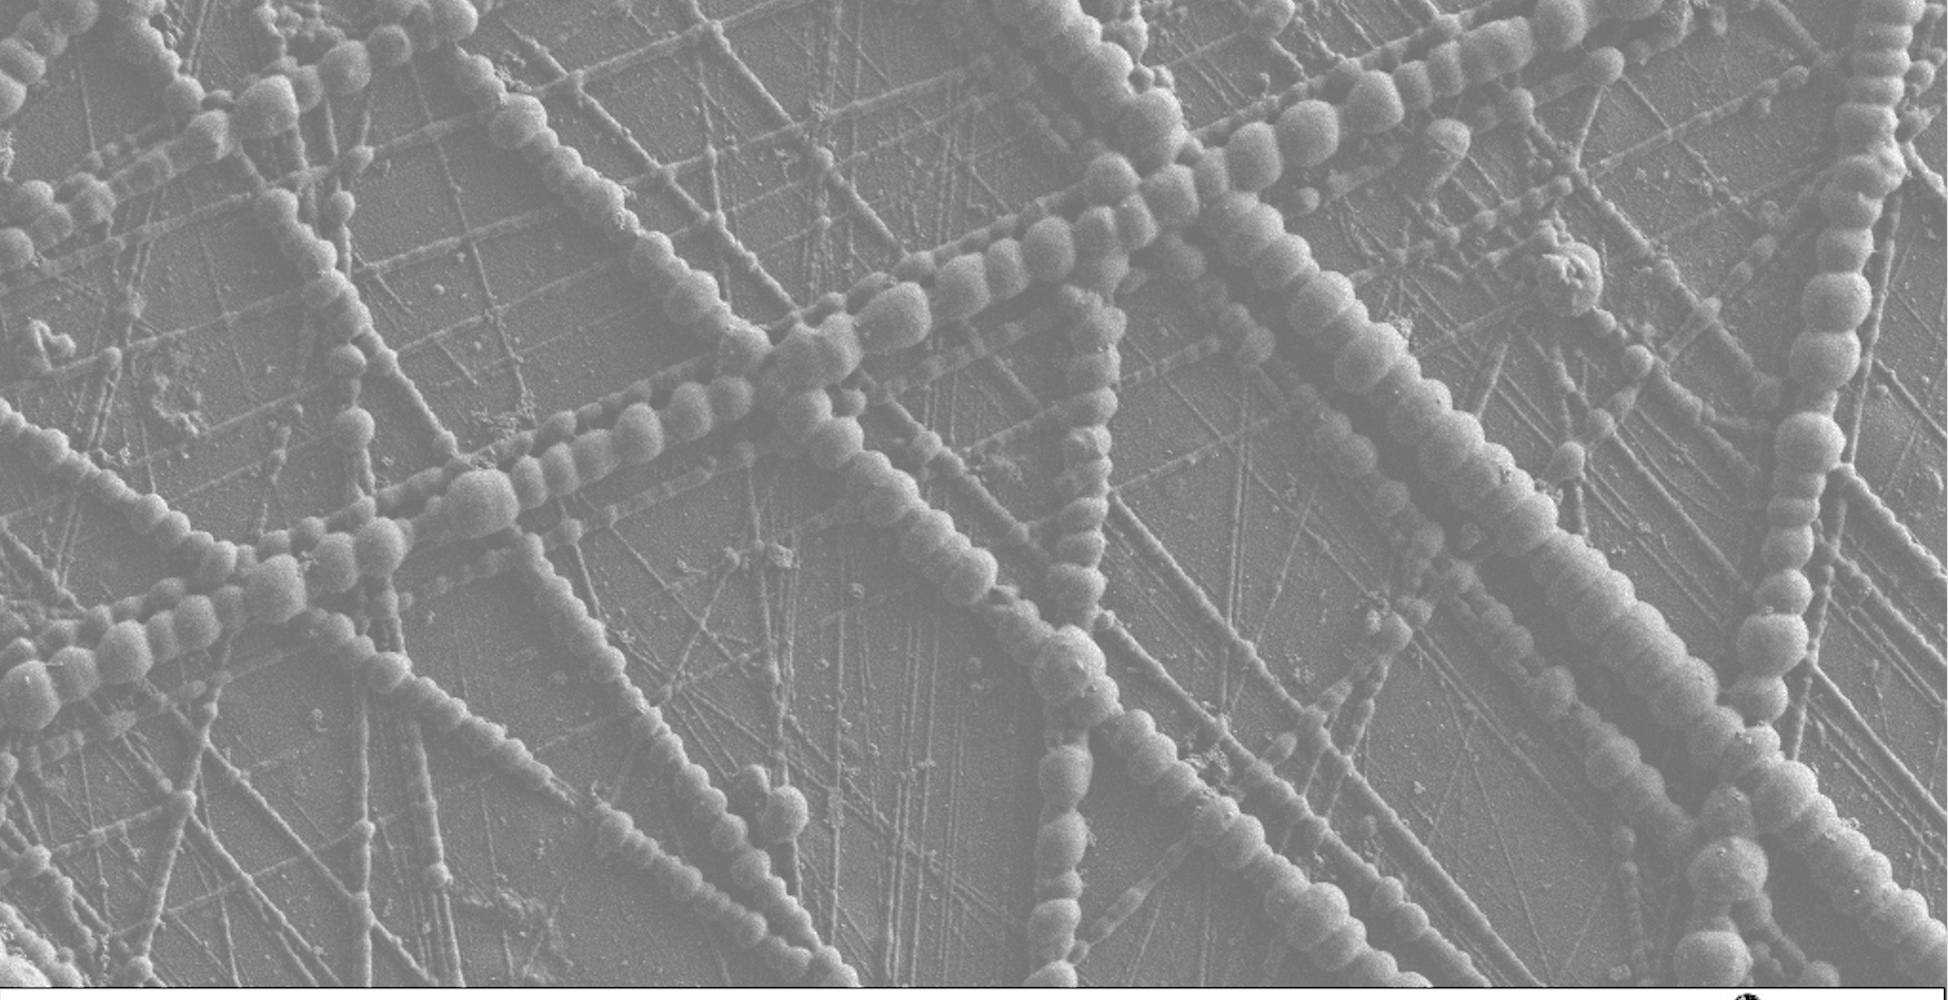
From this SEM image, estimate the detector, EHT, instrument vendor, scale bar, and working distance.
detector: SE2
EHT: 1 kV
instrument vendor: Zeiss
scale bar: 2000 nm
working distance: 3.5 mm